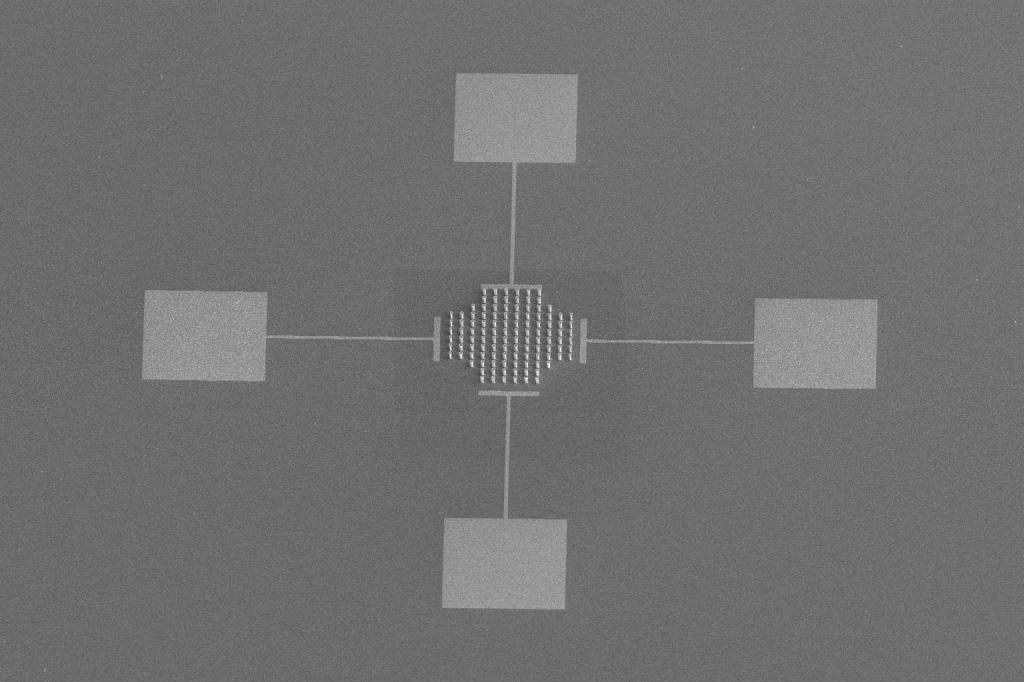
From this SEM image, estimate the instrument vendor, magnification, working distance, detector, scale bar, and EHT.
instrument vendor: FEI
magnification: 0.25 K X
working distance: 21.6 mm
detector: ETD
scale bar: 300000 nm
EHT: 5 kV